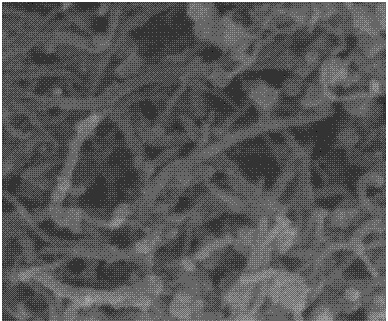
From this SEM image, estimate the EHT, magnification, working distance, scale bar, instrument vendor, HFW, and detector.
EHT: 10 kV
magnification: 200 K X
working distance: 4.8 mm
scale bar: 400 nm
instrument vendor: FEI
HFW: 1.5 µm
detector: TLD